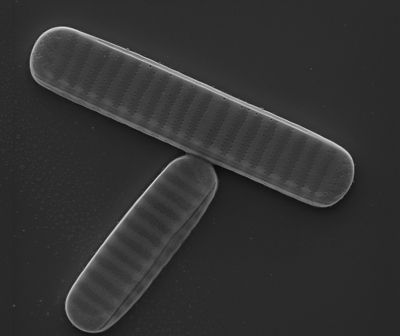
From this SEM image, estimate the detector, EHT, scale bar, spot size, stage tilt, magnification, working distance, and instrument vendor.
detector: SE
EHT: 10 kV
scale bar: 5000 nm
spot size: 3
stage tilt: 0.7°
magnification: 15 K X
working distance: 9.5 mm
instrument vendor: FEI